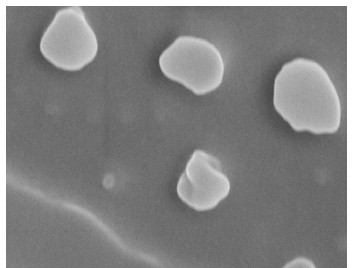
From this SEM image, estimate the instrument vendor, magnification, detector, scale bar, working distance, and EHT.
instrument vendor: Hitachi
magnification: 40 K X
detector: SE(M)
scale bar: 1000 nm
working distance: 6.4 mm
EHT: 15 kV